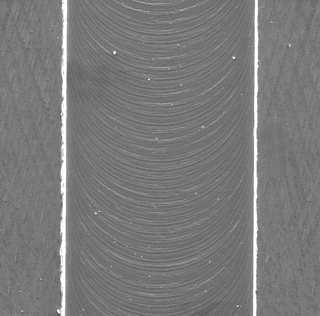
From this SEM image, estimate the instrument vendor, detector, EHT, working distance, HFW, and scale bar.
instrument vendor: Tescan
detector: SE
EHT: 20 kV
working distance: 15.22 mm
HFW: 843 µm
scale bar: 200000 nm